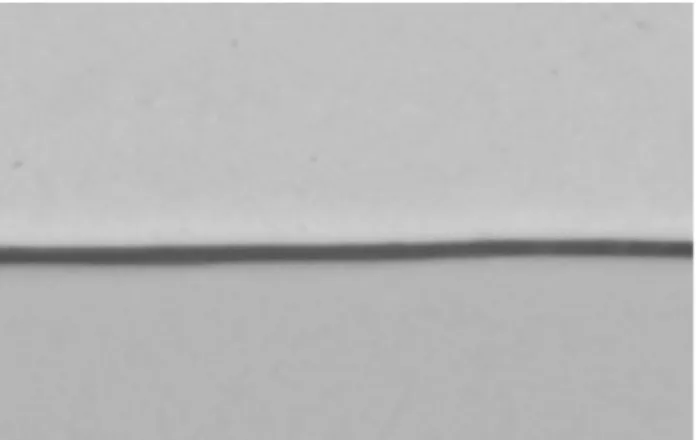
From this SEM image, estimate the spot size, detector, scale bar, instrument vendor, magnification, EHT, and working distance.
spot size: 6.5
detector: BSE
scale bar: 2000 nm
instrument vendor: FEI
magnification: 10 K X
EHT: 20 kV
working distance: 11.7 mm